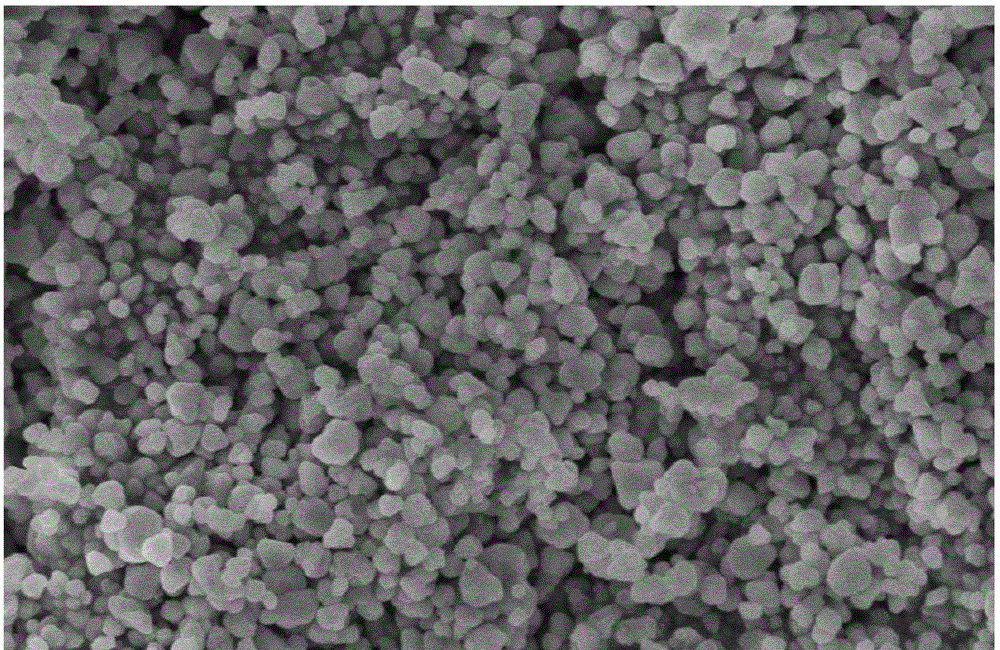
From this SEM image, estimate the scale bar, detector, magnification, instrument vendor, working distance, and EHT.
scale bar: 2000 nm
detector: SE(M)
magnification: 20 K X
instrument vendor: Hitachi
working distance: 12.8 mm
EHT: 5 kV